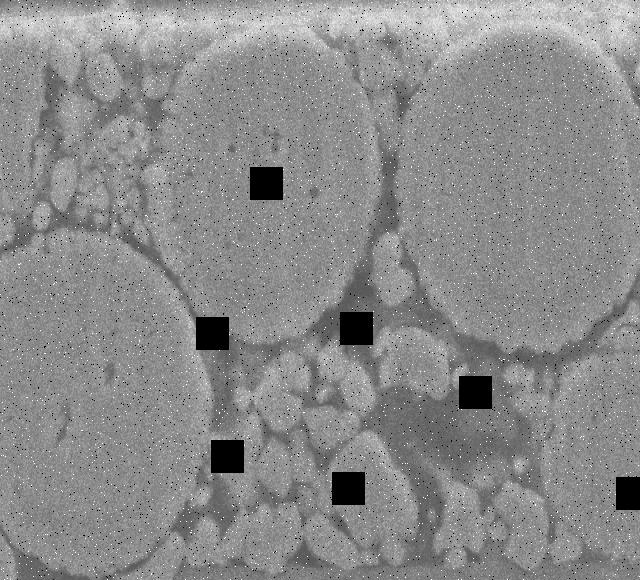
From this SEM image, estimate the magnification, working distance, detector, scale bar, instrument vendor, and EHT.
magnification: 4 K X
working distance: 8 mm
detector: SEI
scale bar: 5000 nm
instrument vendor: JEOL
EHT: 15 kV